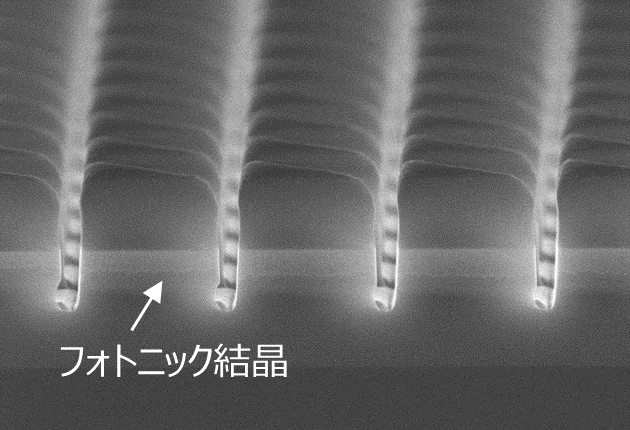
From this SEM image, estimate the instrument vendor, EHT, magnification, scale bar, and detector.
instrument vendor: Hitachi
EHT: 10 kV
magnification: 22 K X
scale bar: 2000 nm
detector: SE